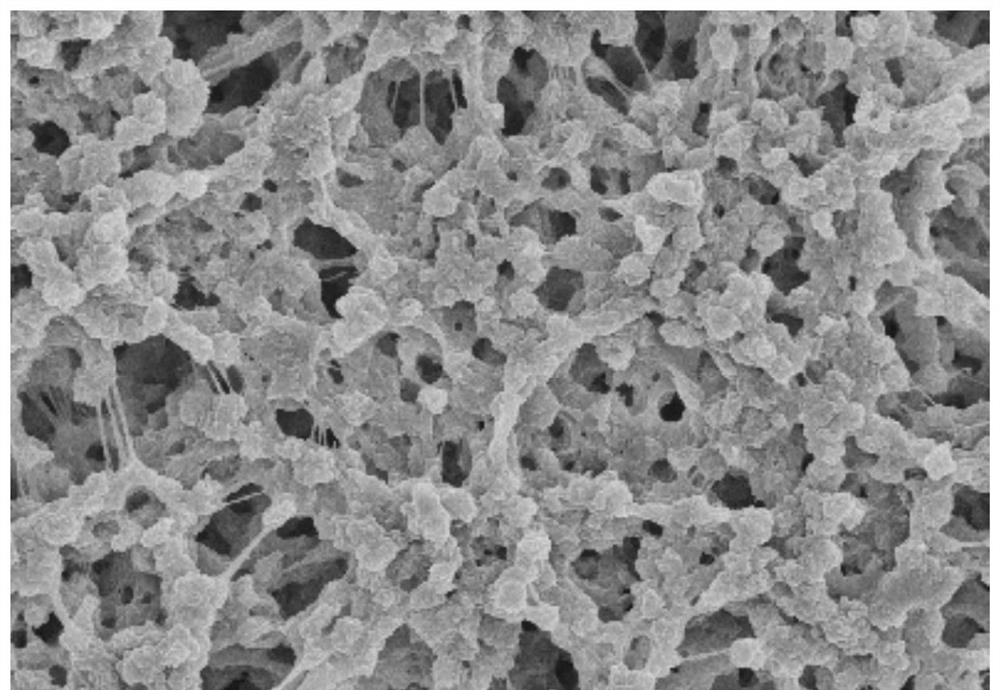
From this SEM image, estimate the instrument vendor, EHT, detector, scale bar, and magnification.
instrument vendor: Hitachi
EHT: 5 kV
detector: SE(M)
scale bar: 5000 nm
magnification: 10 K X